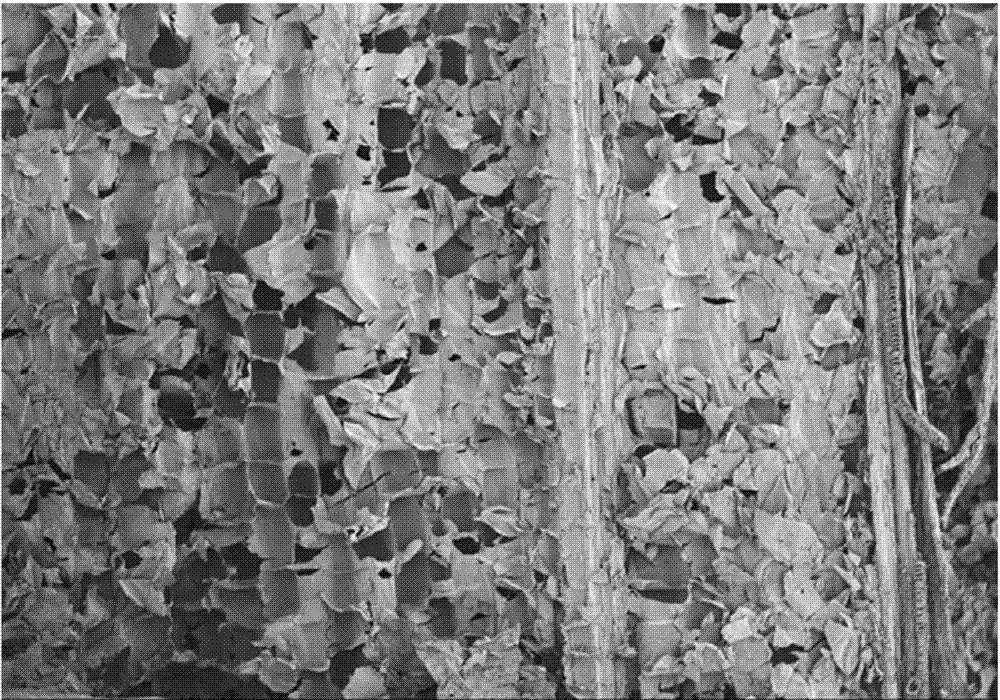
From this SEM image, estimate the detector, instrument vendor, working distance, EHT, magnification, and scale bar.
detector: SE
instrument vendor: Hitachi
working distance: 15.5 mm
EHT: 1 kV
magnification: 0.05 K X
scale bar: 1000000 nm